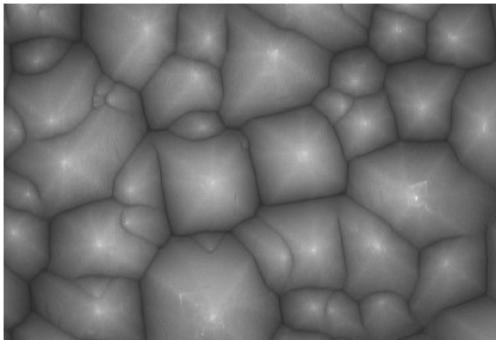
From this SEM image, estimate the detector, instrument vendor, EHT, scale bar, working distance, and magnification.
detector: SE(U)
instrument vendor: Hitachi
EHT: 10 kV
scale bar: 2000 nm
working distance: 8.4 mm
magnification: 20 K X